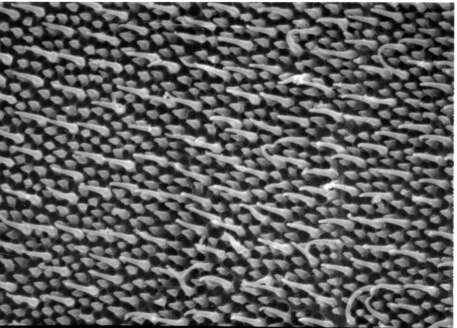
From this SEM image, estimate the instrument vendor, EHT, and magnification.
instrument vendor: JEOL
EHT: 20 kV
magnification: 10 K X